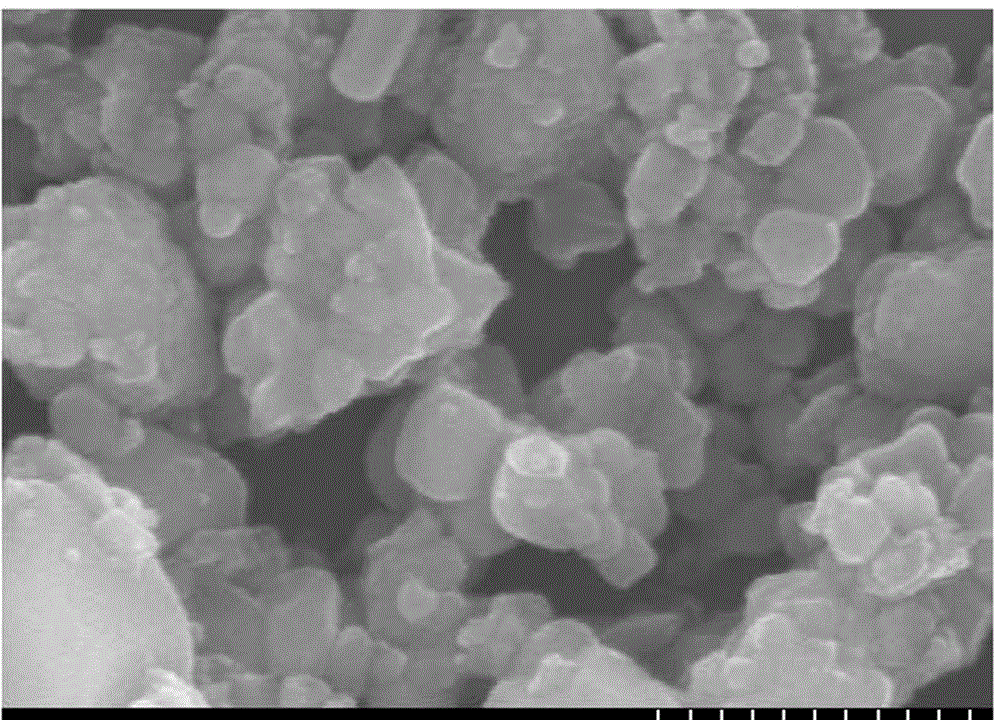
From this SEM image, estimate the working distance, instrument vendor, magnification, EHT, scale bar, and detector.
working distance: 15.5 mm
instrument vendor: Hitachi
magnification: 80 K X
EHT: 15 kV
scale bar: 500 nm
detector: SE(M)